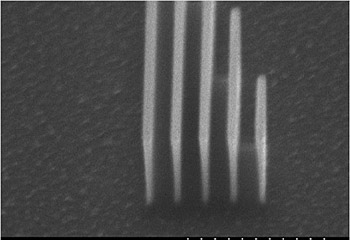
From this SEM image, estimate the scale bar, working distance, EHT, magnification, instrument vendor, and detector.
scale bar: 10000 nm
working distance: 20.8 mm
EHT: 20 kV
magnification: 5 K X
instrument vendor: Hitachi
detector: SE